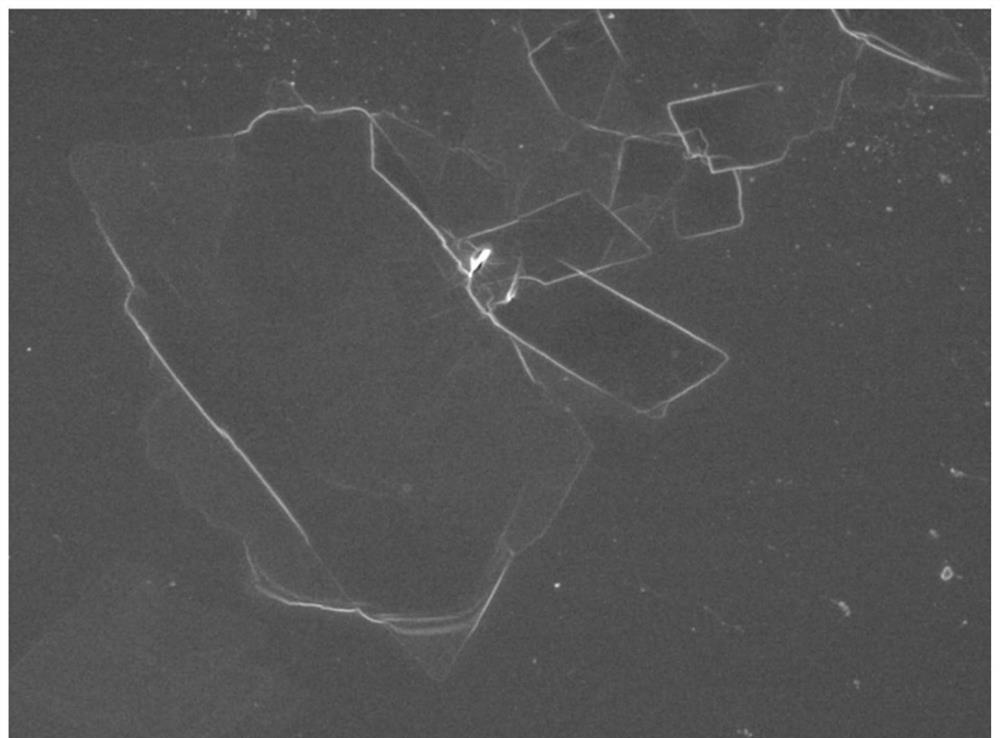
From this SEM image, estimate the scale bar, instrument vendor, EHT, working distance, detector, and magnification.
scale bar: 10000 nm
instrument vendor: JEOL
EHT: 5 kV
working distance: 8.4 mm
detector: SEI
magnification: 2.7 K X